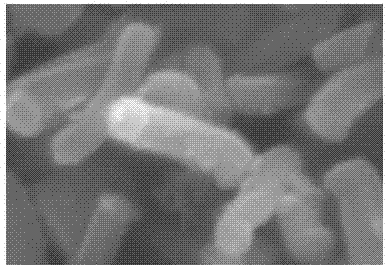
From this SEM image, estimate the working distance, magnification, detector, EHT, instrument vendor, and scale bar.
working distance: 7.7 mm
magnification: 45 K X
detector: SE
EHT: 20 kV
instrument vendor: Hitachi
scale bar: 1000 nm